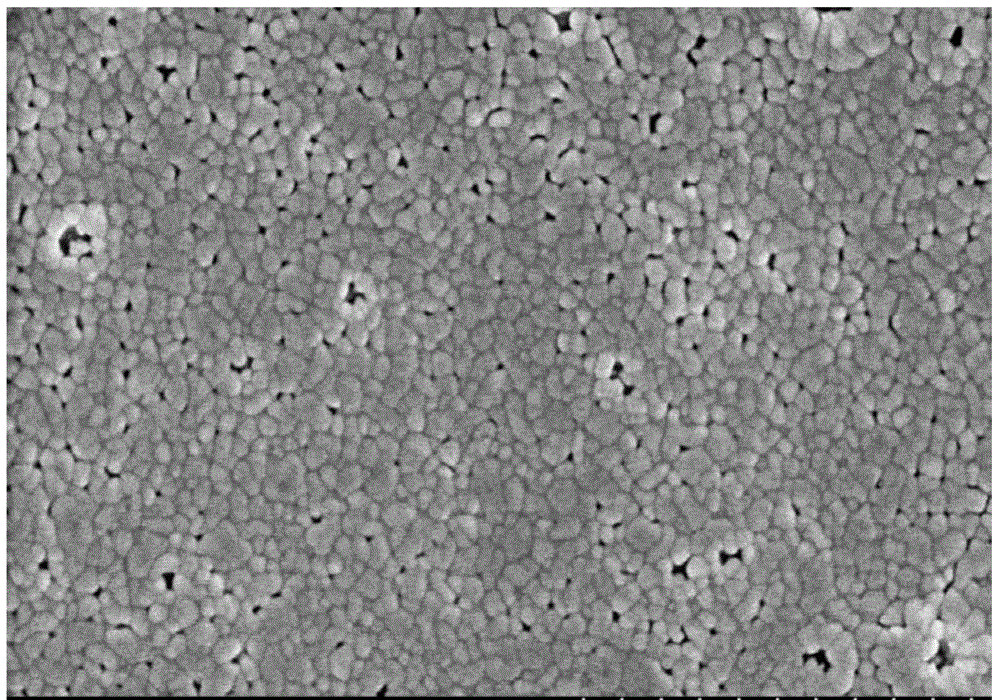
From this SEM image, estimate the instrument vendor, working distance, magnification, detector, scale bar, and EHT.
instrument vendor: Hitachi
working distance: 10.2 mm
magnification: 50 K X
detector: SE(M)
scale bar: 1000 nm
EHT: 3 kV